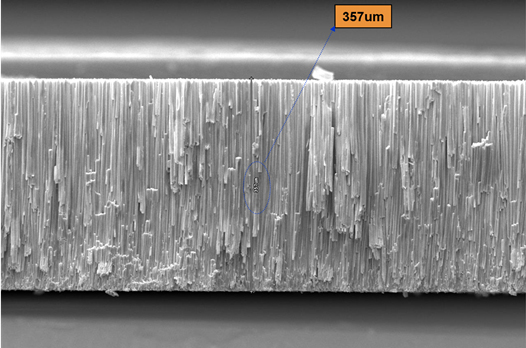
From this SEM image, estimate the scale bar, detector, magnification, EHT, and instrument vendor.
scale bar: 300000 nm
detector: SED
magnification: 0.15 K X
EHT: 30 kV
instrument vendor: other